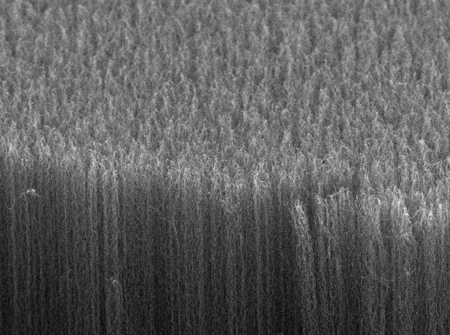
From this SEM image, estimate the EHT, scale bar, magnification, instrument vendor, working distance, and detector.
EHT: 5 kV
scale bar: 10000 nm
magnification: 2.5 K X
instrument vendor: JEOL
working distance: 15.9 mm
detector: SEI